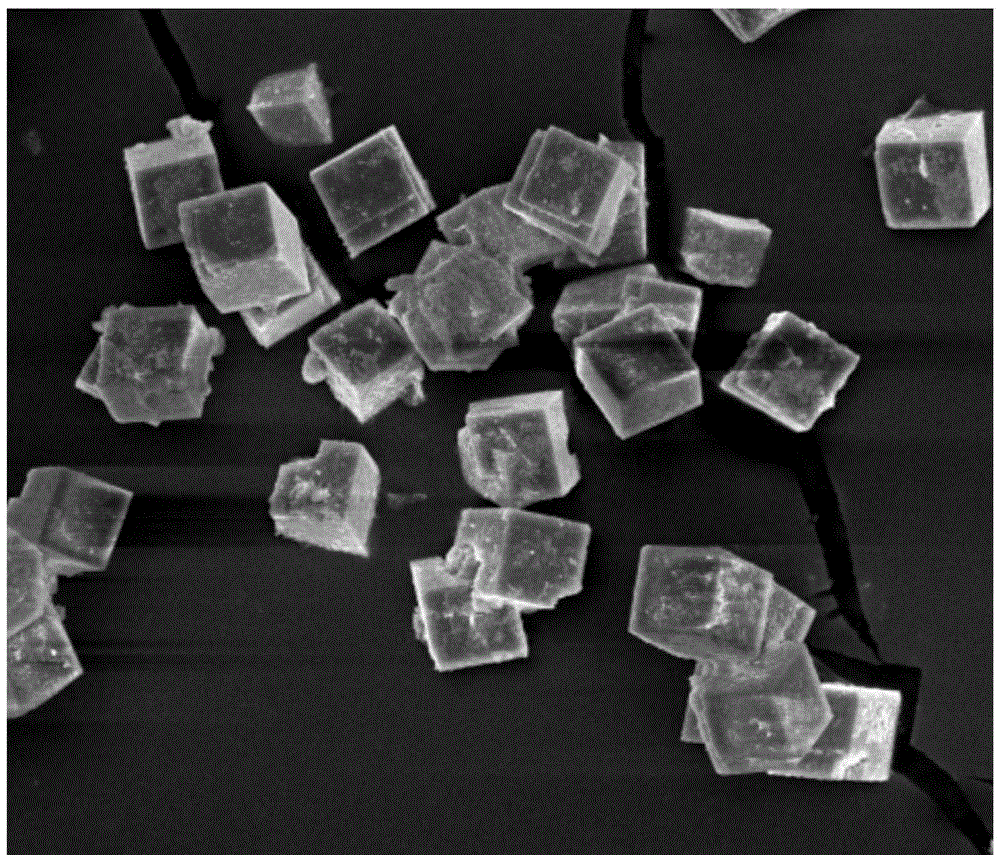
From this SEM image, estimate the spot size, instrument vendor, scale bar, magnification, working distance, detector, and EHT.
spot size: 2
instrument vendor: FEI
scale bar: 10000 nm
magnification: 10 K X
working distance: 5.6 mm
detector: TLD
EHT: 5 kV